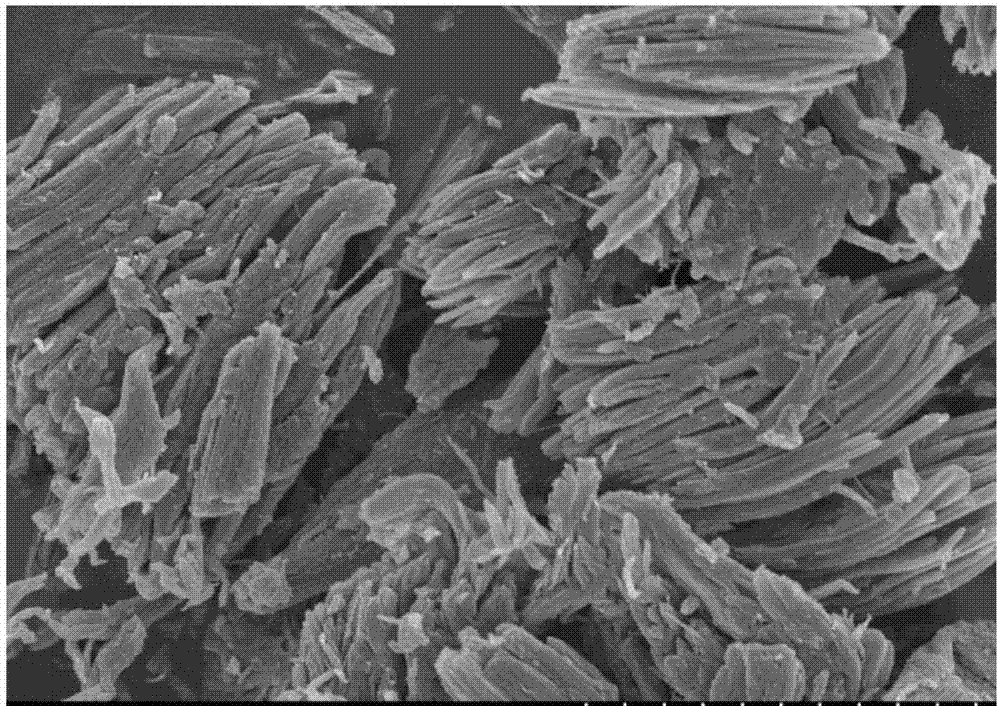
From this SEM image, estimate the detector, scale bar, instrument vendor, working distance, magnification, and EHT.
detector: SE(M)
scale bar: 5000 nm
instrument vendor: Hitachi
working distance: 7.1 mm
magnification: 10 K X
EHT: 5 kV